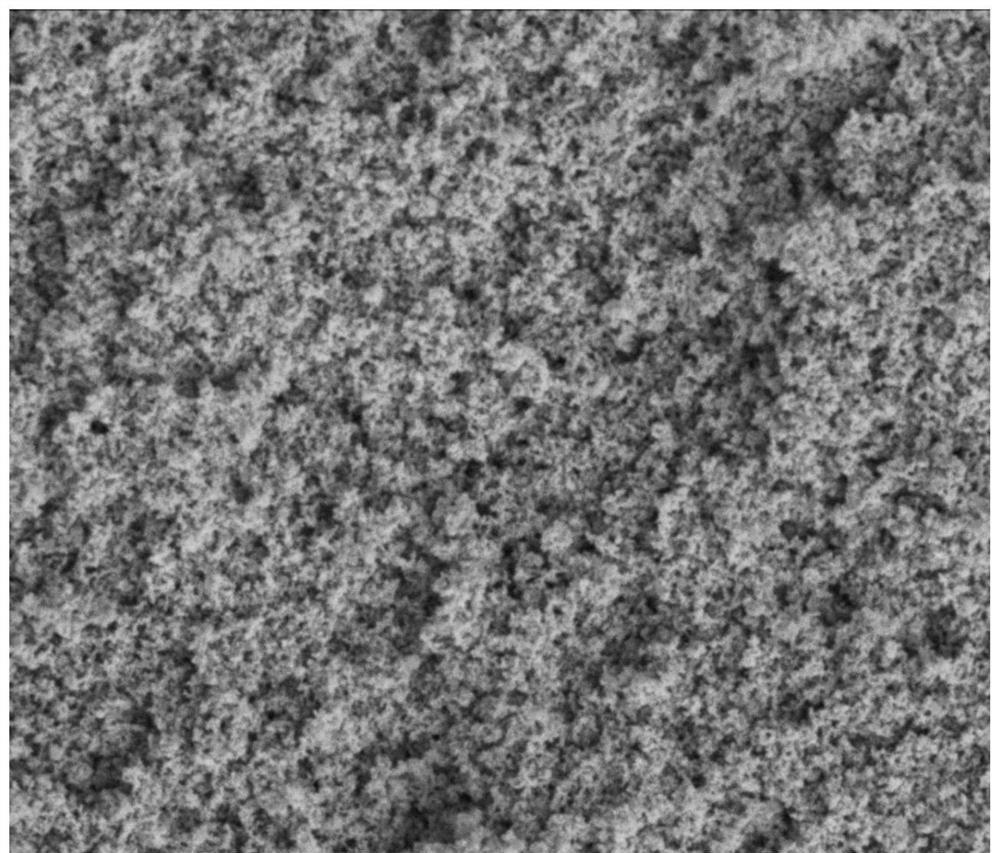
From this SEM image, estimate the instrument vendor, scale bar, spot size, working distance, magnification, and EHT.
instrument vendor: FEI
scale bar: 4000 nm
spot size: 3.5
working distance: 5.7 mm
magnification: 20 K X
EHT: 3 kV